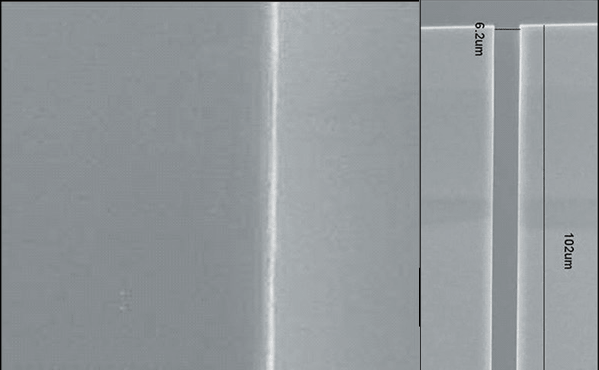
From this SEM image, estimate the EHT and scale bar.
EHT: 7.5 kV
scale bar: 50000 nm